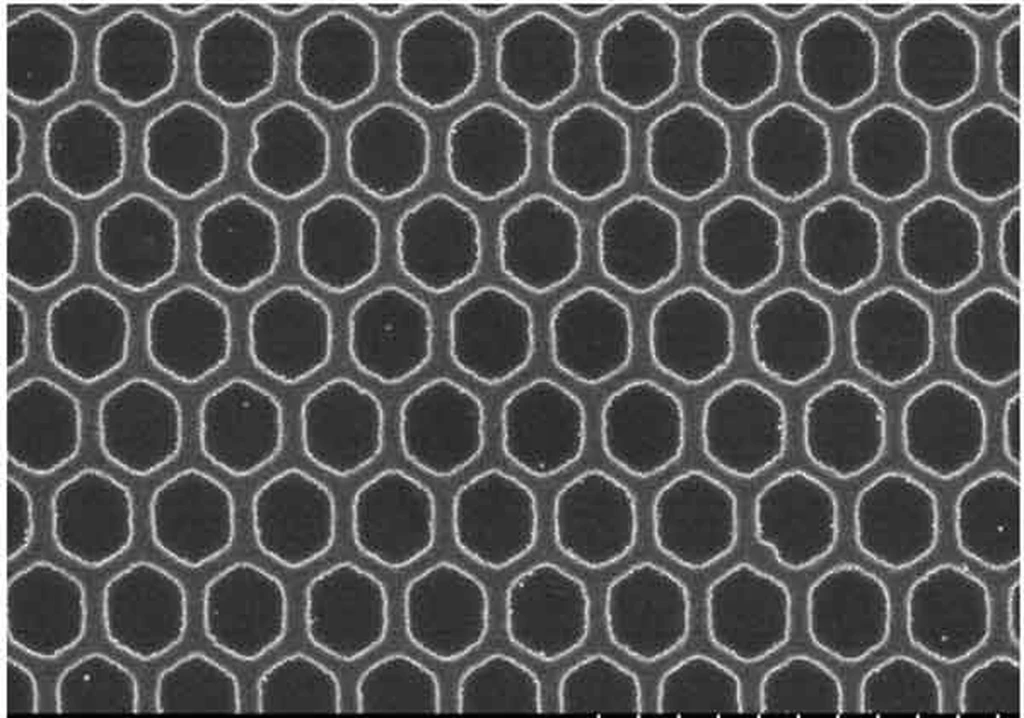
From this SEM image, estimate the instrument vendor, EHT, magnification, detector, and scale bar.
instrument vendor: Hitachi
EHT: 10 kV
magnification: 0.5 K X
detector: SE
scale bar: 100000 nm